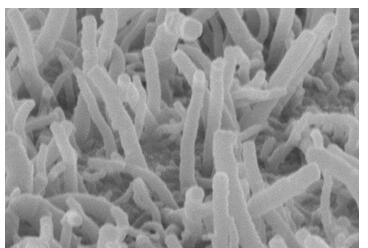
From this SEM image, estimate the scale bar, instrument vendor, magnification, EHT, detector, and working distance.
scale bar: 1000 nm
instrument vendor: Hitachi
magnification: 50 K X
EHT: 5 kV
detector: SE(M)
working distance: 10.9 mm